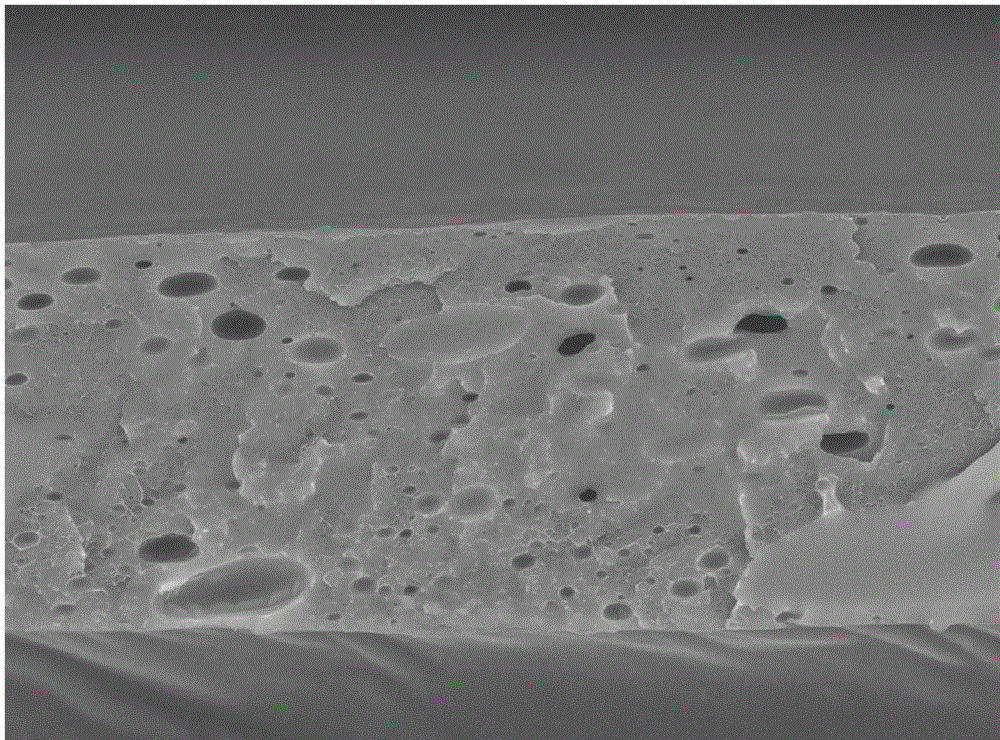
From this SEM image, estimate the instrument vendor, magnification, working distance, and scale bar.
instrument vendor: Hitachi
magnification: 1.5 K X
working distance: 4.6 mm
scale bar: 50000 nm